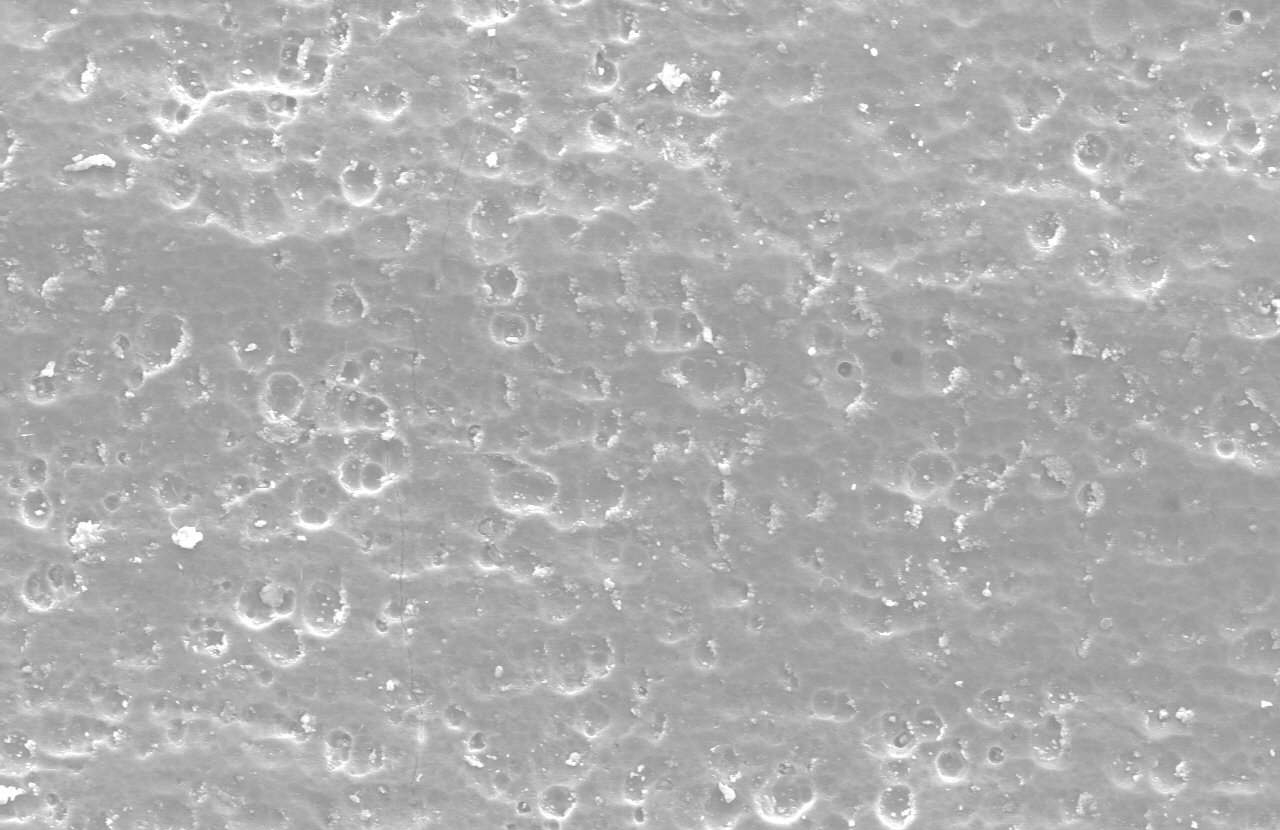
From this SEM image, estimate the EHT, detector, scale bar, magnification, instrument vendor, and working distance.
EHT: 15 kV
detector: SE(M)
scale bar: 100000 nm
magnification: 0.5 K X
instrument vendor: Hitachi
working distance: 17.5 mm